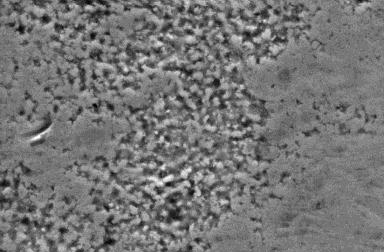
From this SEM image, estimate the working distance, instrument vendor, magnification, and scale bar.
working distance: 8.4 mm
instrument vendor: FEI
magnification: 1.6 K X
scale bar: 20000 nm